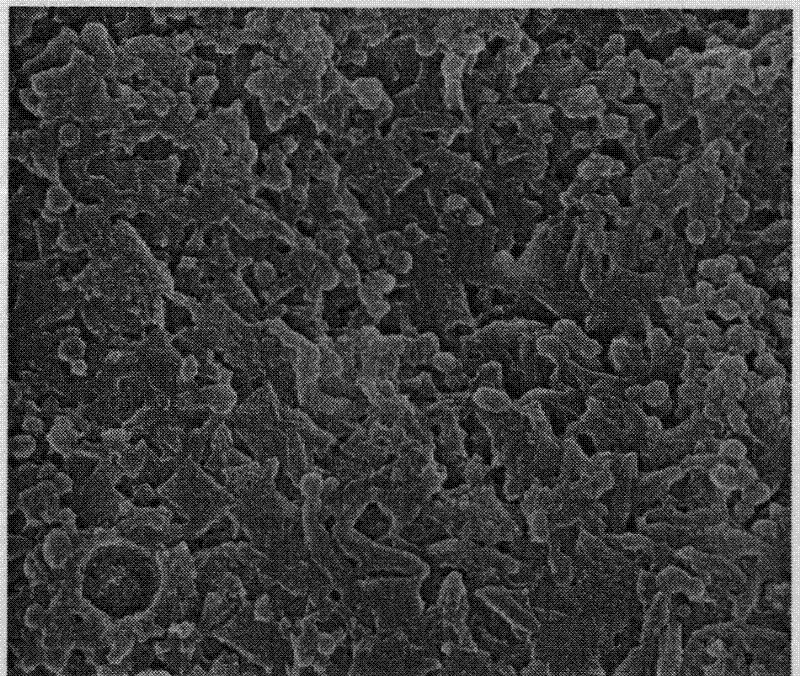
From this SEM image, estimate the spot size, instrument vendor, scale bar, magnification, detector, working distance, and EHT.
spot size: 3.5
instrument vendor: FEI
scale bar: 5000 nm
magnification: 10 K X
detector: ETD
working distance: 10.8 mm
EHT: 25 kV